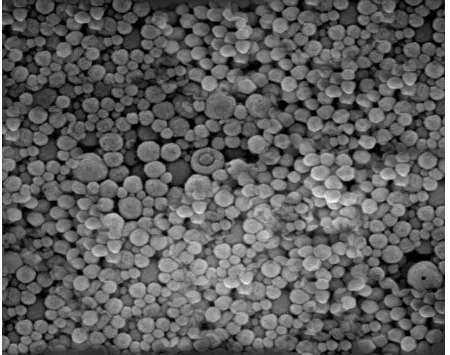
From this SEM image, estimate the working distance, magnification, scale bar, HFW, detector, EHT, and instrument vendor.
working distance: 15.46 mm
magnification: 48.9 K X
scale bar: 500 nm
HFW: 2.83 µm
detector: SE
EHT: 5 kV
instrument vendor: Tescan